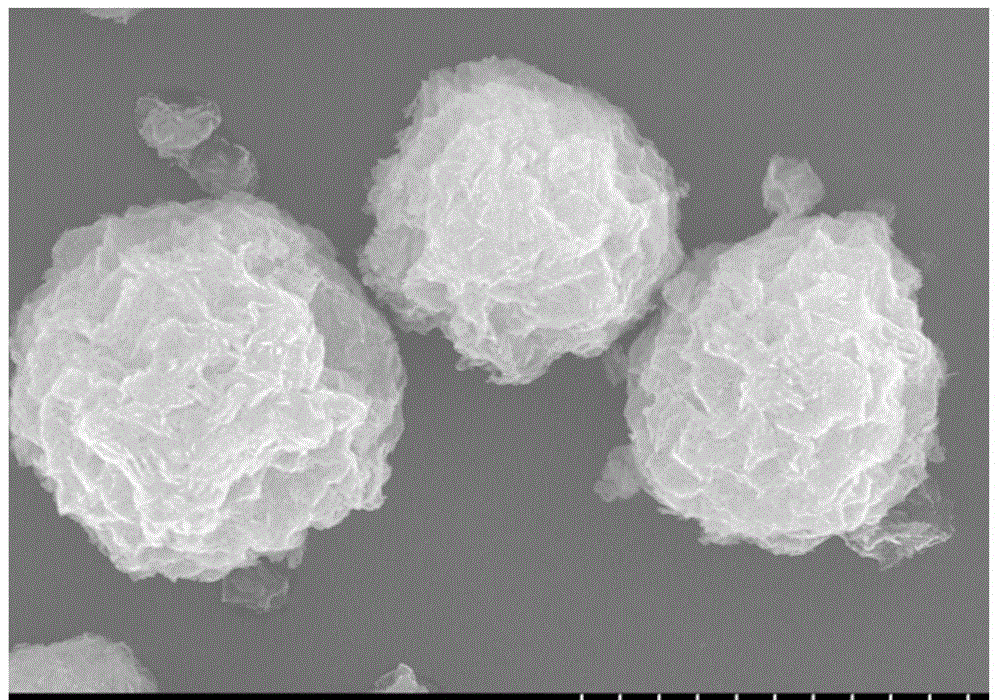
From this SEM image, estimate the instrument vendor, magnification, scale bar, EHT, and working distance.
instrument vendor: Hitachi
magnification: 5 K X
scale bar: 10000 nm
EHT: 20 kV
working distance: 11.6 mm